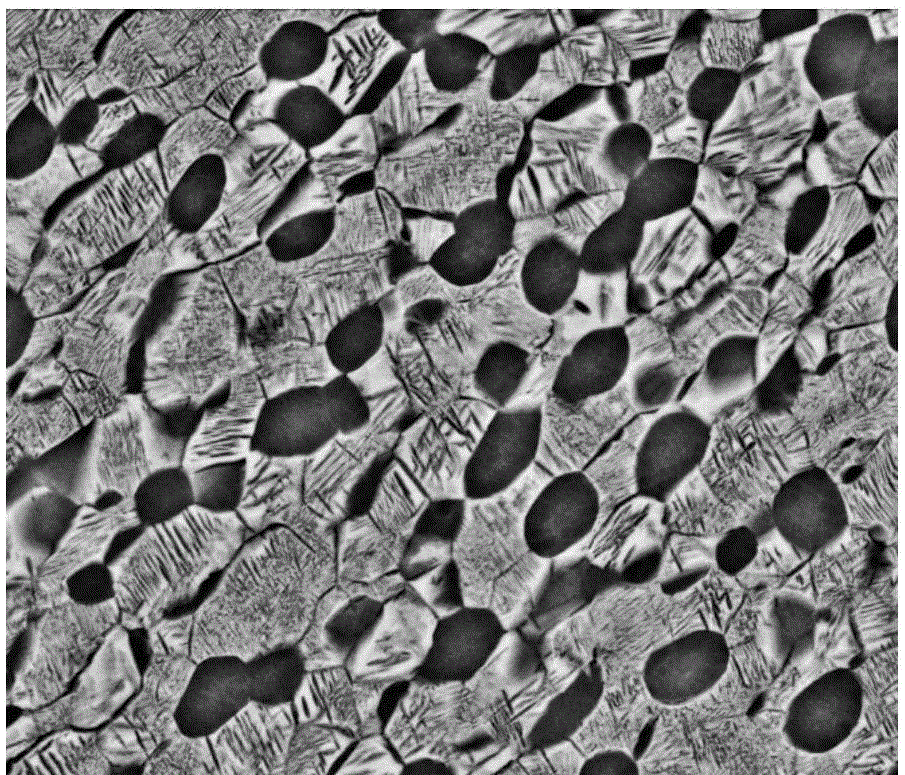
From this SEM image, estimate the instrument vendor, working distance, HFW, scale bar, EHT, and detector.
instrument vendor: FEI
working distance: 9.6 mm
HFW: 29.8 µm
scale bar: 5000 nm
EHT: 20 kV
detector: BSED